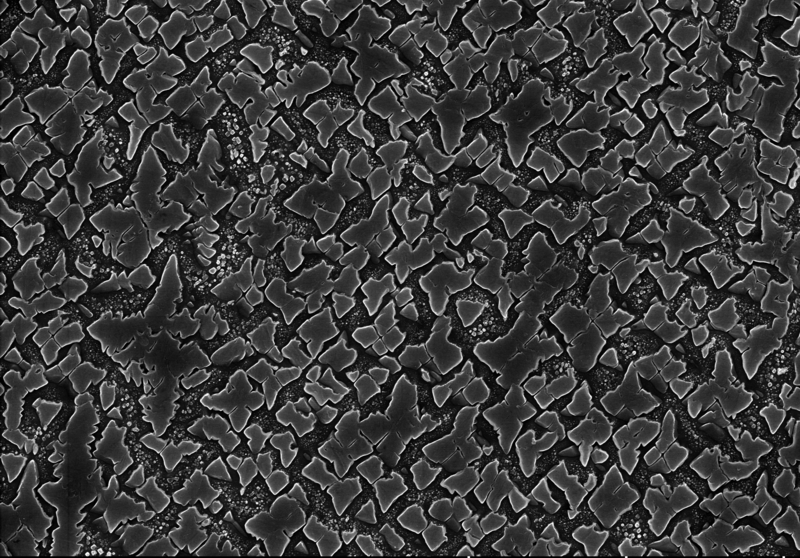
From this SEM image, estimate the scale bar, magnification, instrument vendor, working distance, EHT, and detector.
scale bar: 5000 nm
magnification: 10 K X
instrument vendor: Hitachi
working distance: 10.3 mm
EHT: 15 kV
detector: SE(U)